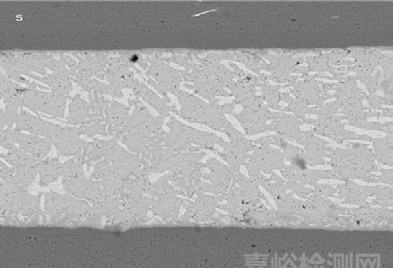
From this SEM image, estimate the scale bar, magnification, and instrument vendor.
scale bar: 20000 nm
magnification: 2 K X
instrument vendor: other